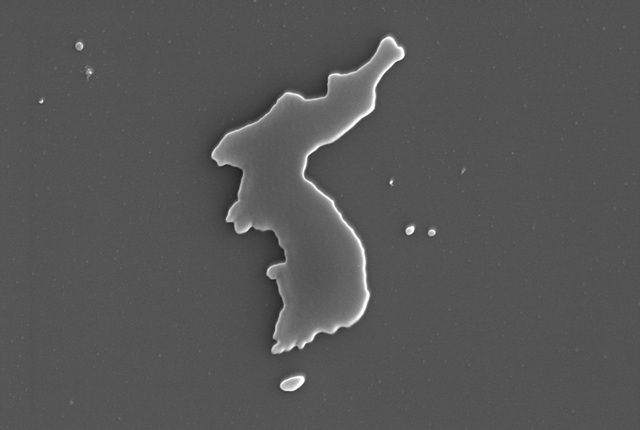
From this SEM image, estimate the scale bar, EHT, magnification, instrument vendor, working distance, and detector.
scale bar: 10000 nm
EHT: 15 kV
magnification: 5 K X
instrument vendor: JEOL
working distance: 14.9 mm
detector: SE